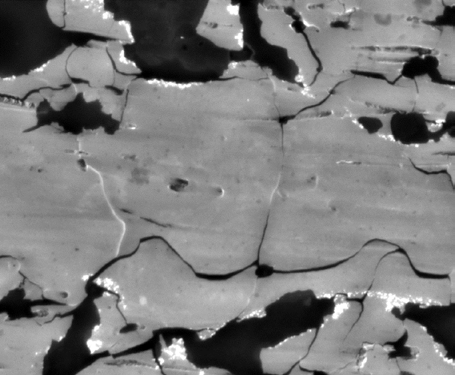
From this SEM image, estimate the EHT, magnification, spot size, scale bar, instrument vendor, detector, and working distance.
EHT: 30 kV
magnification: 1 K X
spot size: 5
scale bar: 50000 nm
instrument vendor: FEI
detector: SSD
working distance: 9.3 mm